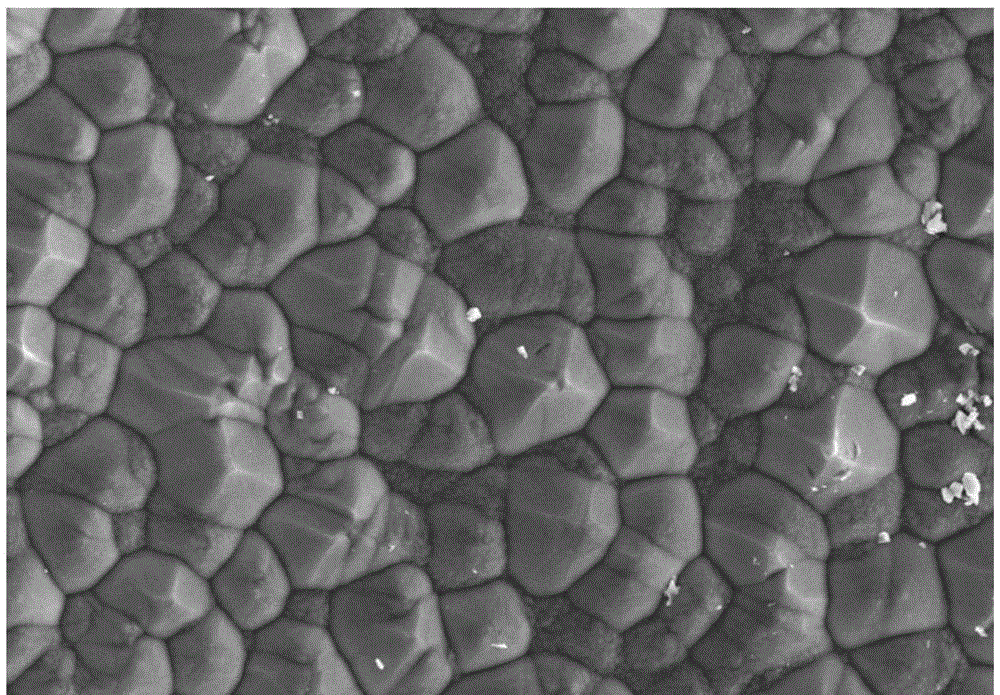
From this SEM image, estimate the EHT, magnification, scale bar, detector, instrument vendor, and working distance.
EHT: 10 kV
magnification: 2 K X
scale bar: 20000 nm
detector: SE(M)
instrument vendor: Hitachi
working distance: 8 mm